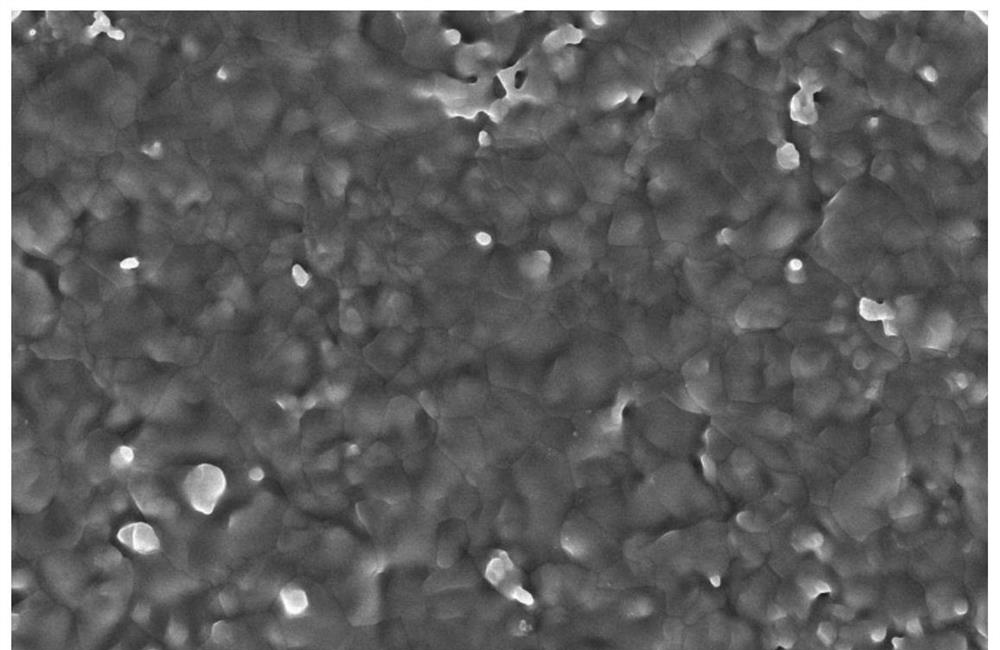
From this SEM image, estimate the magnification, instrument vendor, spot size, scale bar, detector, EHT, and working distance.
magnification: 10 K X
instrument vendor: FEI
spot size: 8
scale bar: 10000 nm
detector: ETD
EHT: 20 kV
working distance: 9.5 mm